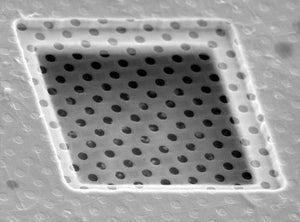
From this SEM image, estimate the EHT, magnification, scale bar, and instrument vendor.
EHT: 25 kV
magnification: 2 K X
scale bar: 10000 nm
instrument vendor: other